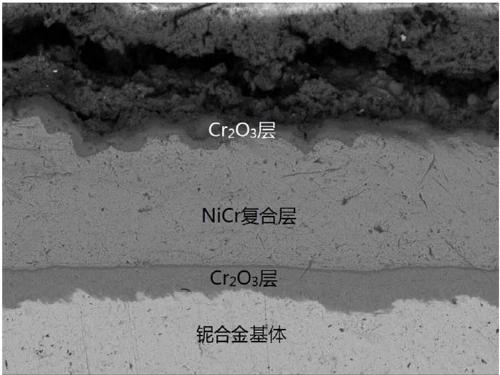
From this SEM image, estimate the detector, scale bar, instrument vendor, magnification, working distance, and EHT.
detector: COMPO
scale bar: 1000 nm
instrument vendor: JEOL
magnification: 5 K X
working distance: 9.3 mm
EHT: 5 kV